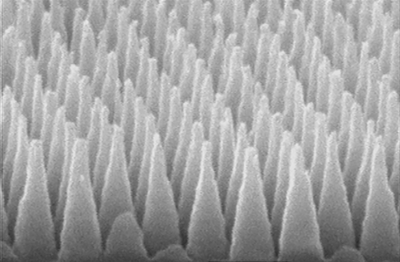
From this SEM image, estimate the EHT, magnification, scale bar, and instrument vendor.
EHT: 15 kV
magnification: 50 K X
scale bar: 500 nm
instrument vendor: JEOL